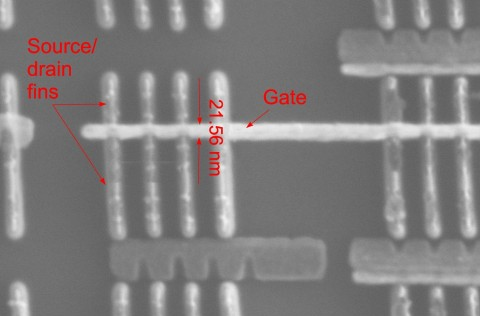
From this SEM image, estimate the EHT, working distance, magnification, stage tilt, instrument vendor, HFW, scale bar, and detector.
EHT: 30 kV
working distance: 3.6 mm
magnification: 250 K X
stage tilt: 0°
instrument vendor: FEI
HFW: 0.829 µm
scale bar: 200 nm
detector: ETD